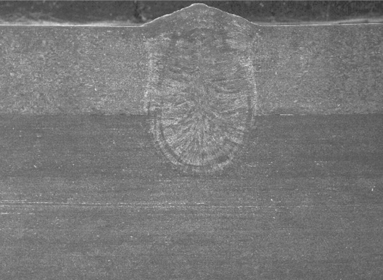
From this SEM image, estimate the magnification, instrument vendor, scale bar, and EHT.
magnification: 0.027 K X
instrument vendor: JEOL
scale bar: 500000 nm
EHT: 22 kV